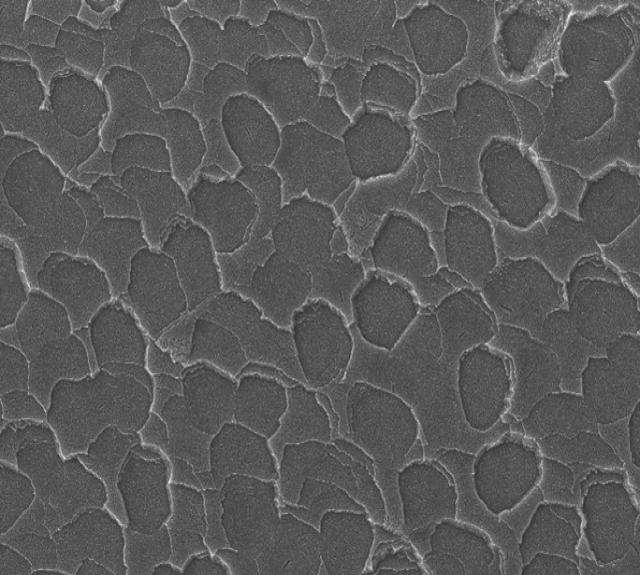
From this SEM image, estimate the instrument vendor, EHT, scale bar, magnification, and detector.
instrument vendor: JEOL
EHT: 30 kV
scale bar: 50000 nm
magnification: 0.33 K X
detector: SEI